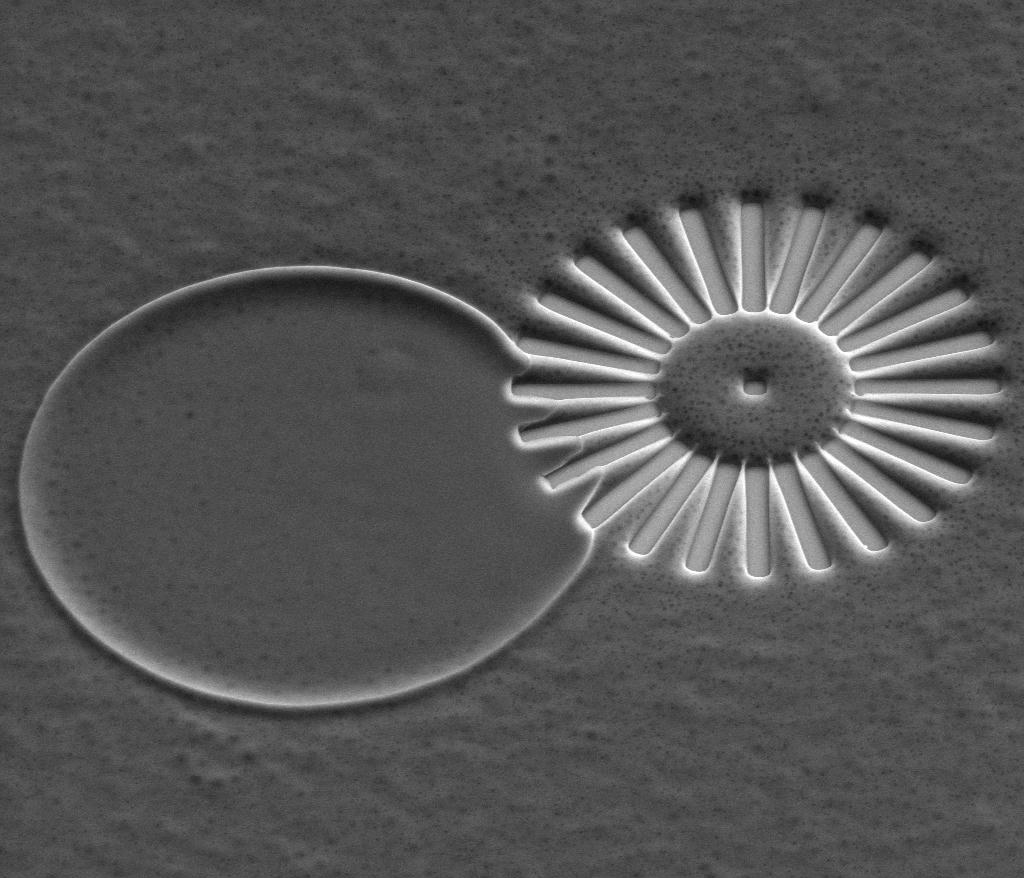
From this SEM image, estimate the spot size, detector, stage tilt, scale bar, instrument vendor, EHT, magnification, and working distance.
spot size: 4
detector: LFD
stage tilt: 40°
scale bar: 100000 nm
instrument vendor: FEI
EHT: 15 kV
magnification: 0.524 K X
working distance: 14 mm